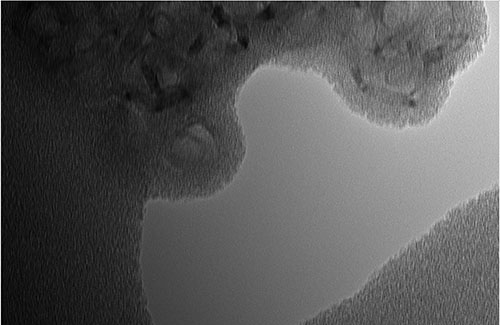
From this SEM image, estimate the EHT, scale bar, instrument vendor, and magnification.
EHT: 120 kV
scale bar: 20 nm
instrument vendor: other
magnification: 250 K X